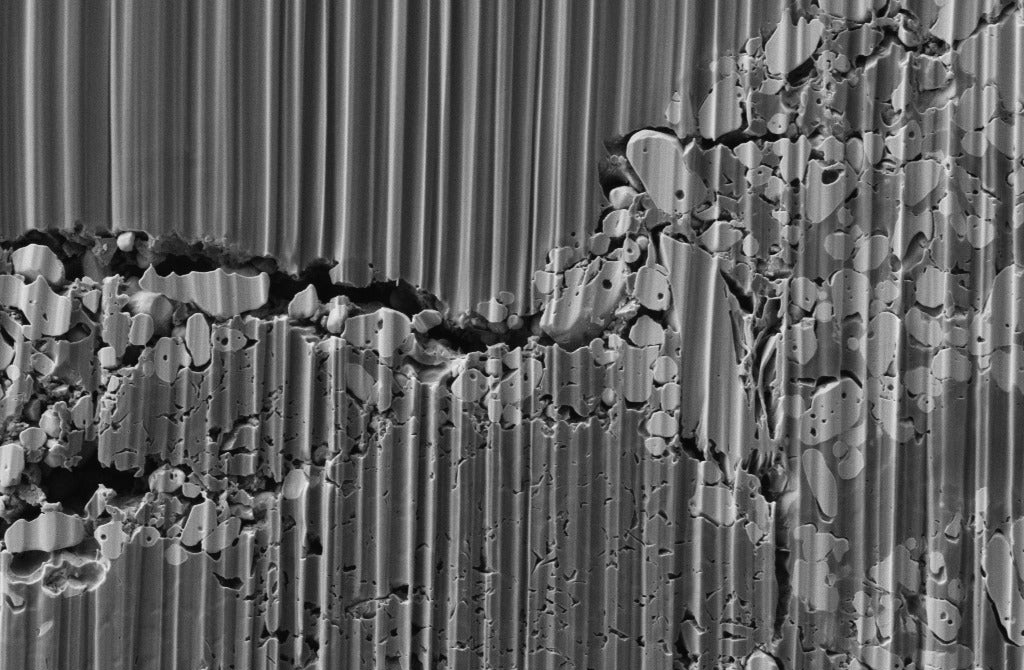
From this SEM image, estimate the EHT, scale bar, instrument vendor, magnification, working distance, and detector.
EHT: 1 kV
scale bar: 1000 nm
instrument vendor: Zeiss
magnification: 10 K X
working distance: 3.7 mm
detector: SE2